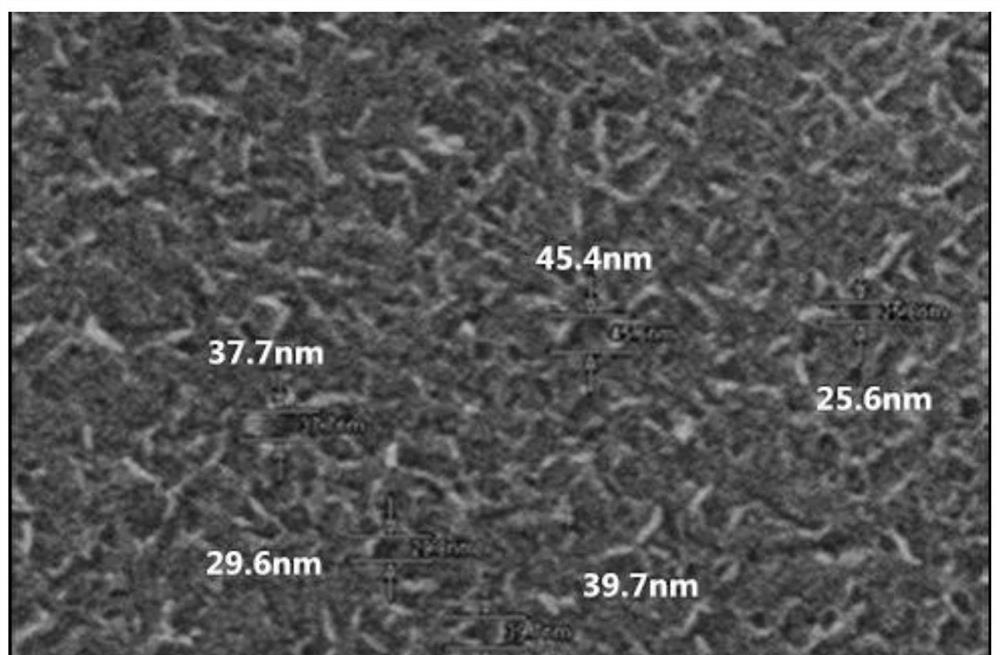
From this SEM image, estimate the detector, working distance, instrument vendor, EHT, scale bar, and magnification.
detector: SE(U)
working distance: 8.2 mm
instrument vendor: Hitachi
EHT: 15 kV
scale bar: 500 nm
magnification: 100 K X